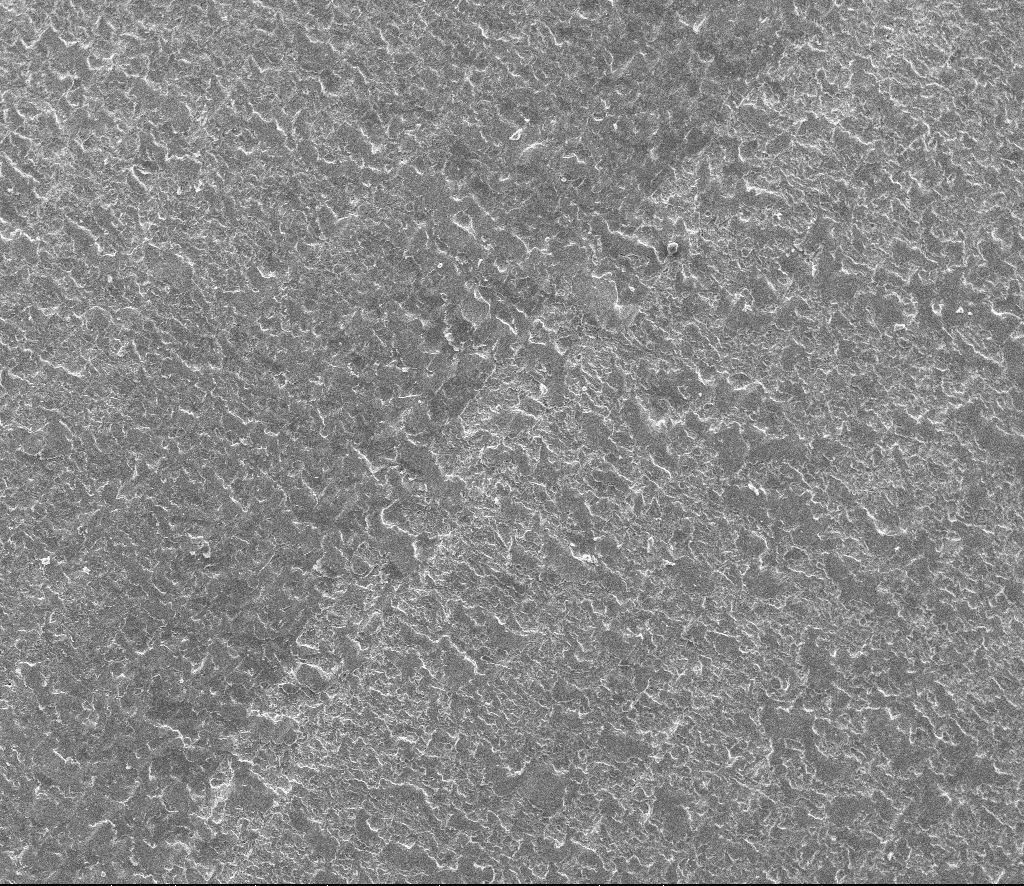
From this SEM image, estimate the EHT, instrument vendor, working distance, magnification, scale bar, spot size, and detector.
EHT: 20 kV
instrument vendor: FEI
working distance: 11.6 mm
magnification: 0.1 K X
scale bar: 500000 nm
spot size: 2.5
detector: ETD